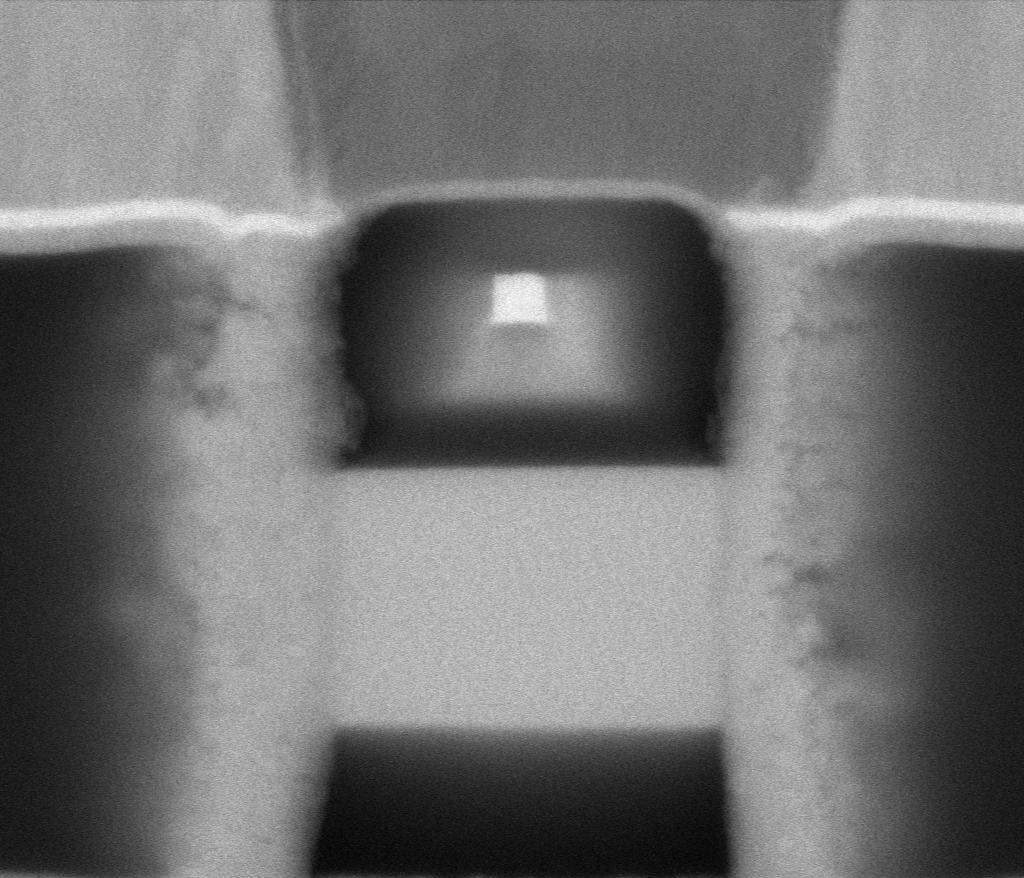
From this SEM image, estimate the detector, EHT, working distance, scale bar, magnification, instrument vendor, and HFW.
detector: MD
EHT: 5 kV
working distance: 2 mm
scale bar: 100 nm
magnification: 568.609 K X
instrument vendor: Thermo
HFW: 0.635 µm